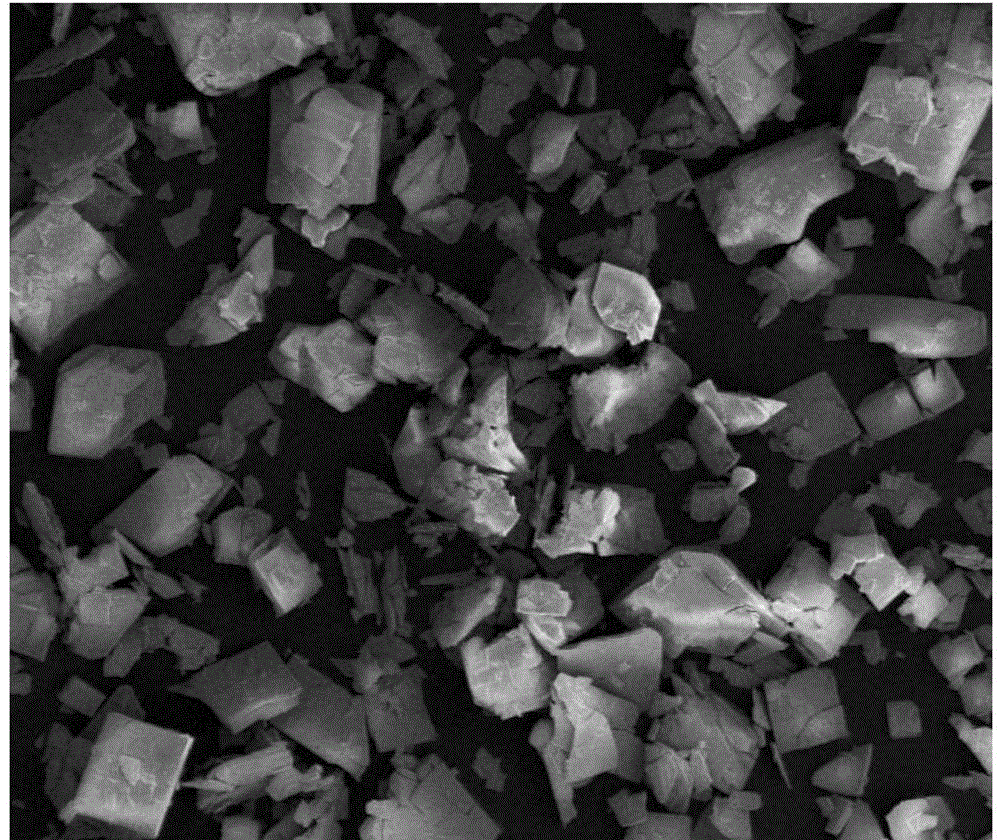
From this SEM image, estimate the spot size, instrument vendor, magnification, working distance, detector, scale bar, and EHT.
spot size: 2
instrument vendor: FEI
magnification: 0.5 K X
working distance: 3 mm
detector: ETD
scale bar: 100000 nm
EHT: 3 kV